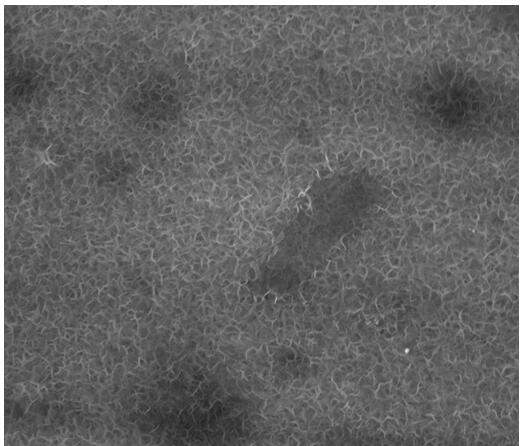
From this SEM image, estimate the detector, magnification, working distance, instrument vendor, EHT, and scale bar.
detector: TLD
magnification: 50 K X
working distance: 4.9 mm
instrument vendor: FEI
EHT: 10 kV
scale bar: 1000 nm